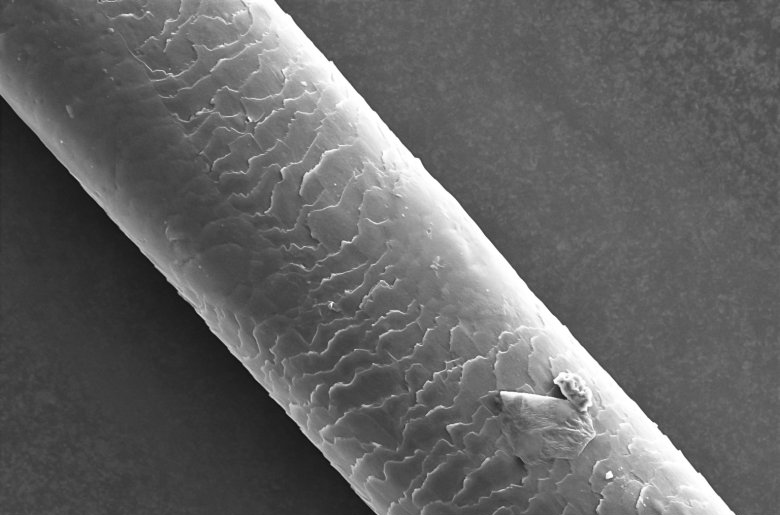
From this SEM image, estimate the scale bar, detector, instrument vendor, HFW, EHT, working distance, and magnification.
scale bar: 50000 nm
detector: ETD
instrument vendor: Thermo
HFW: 254 µm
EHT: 15 kV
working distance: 8.1 mm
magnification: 0.5 K X